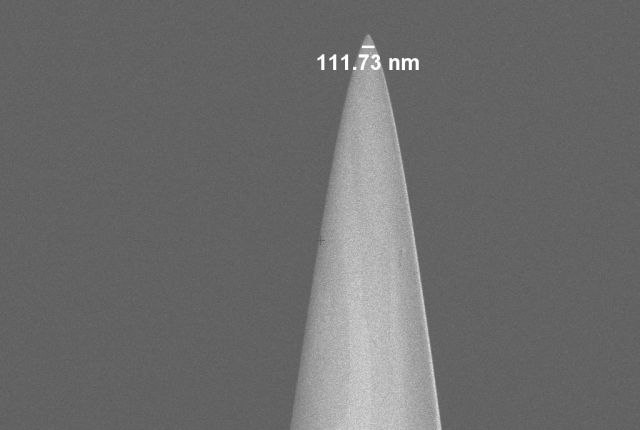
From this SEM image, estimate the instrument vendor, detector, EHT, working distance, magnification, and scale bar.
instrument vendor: Zeiss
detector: InLens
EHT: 2 kV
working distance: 4.6 mm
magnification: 20 K X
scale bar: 2000 nm